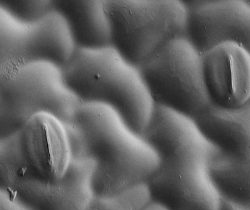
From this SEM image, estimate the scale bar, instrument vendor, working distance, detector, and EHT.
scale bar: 20000 nm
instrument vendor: Zeiss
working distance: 8.1 mm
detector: SE2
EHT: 2 kV